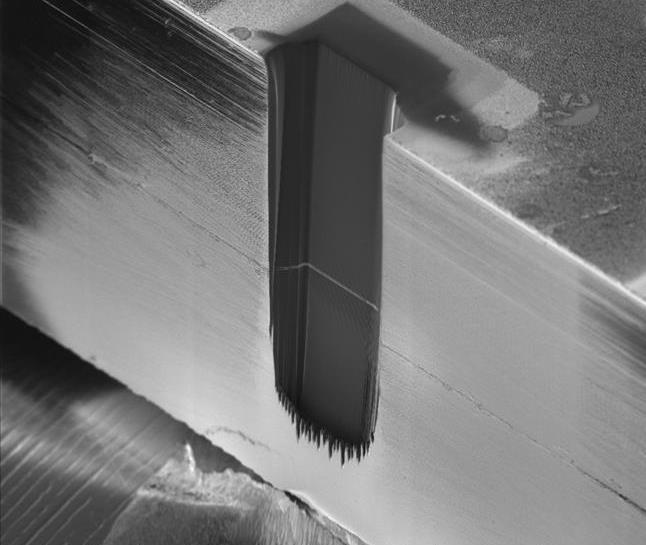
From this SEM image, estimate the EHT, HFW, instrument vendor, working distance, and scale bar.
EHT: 30 kV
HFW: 1000 µm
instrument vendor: FEI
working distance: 16.4 mm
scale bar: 200000 nm